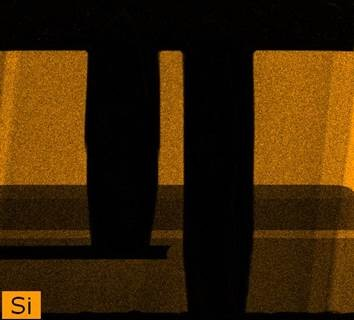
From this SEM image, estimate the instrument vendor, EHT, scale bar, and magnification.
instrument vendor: FEI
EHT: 300 kV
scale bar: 2000 nm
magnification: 10 K X